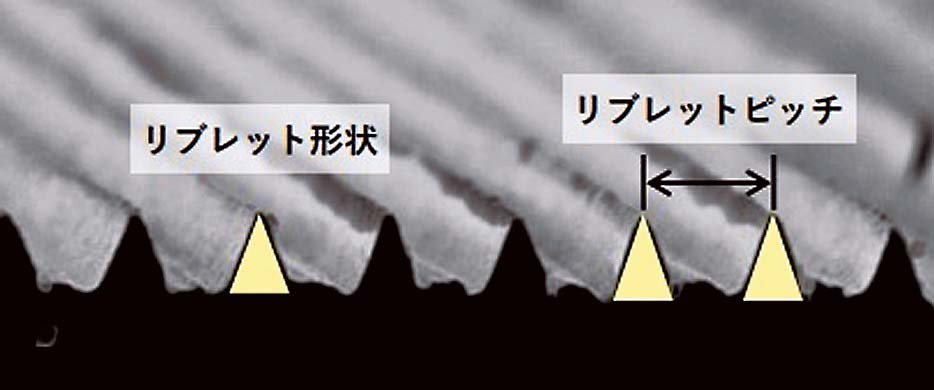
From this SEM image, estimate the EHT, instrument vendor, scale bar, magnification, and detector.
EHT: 15 kV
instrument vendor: JEOL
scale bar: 100000 nm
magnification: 0.2 K X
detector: SED